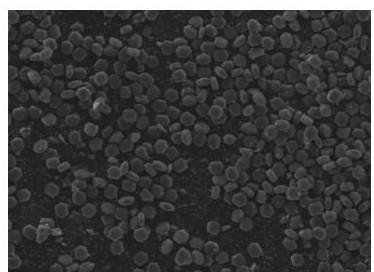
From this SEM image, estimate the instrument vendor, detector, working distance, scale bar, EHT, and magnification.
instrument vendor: JEOL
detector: LED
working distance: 10.4 mm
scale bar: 10000 nm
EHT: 10 kV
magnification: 1.3 K X